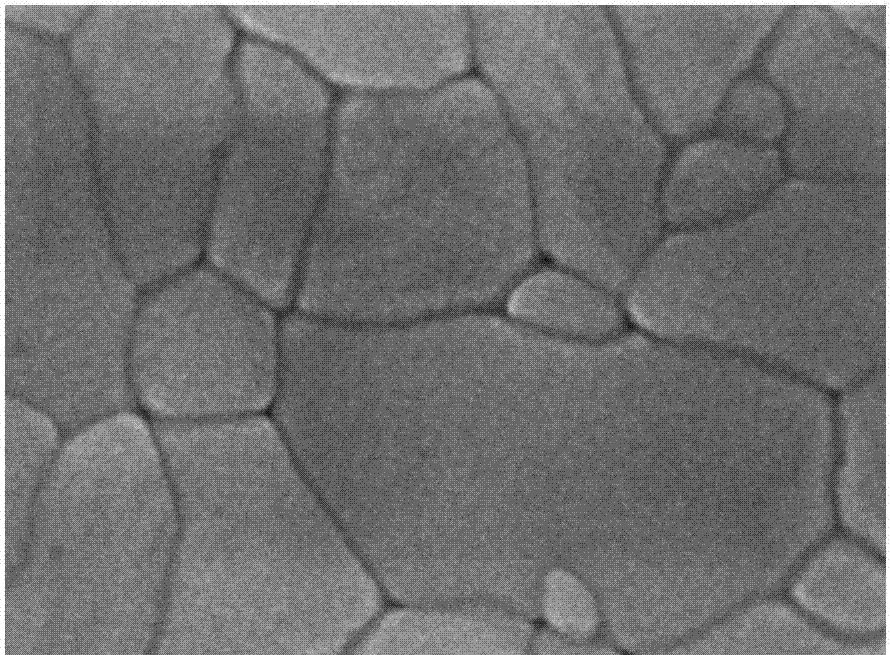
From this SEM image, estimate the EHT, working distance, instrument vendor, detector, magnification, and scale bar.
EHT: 15 kV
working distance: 7.6 mm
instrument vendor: JEOL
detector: SEI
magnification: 80 K X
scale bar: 100 nm